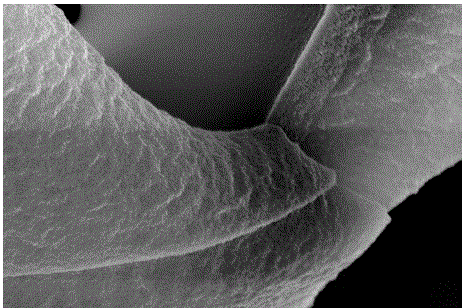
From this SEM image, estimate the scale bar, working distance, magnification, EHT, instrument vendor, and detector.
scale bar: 100 nm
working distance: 6.6 mm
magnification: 50 K X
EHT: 5 kV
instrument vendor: Zeiss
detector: InLens